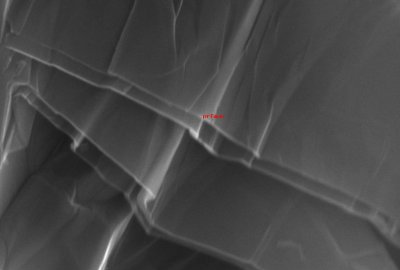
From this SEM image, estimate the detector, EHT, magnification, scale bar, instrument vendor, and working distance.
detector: ETD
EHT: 28 kV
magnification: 28 K X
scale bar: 5000 nm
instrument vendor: FEI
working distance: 10 mm